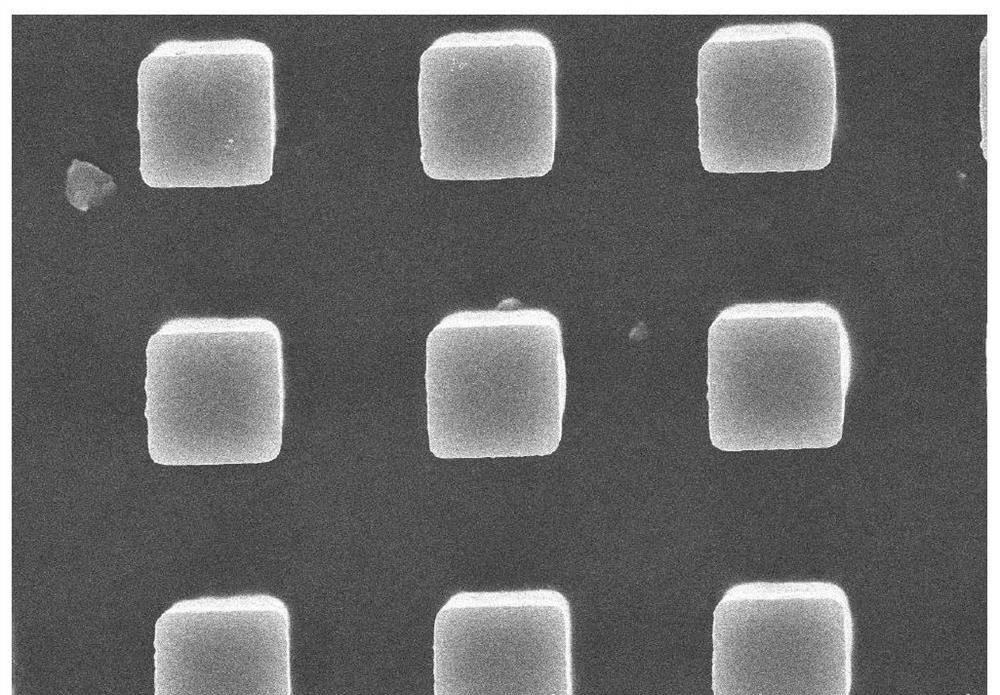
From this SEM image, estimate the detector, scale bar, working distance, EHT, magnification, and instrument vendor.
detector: SE(U)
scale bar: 30000 nm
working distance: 10 mm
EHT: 15 kV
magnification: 1.8 K X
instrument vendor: Hitachi